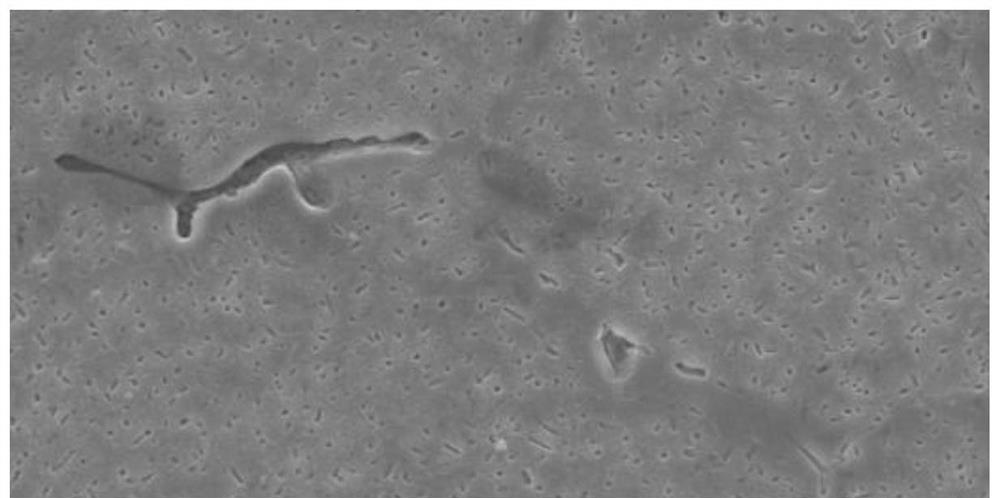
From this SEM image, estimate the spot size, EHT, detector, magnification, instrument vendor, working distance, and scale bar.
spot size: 4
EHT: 15 kV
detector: SE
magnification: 1 K X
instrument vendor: FEI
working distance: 18 mm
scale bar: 10000 nm